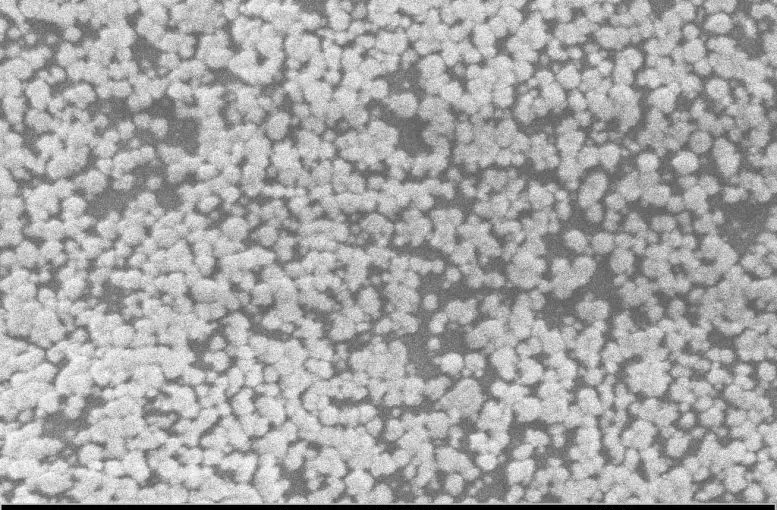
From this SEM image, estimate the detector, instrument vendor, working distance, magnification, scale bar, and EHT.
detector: SE2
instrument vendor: Zeiss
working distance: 8.6 mm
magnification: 32.49 K X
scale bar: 2000 nm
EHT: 5 kV